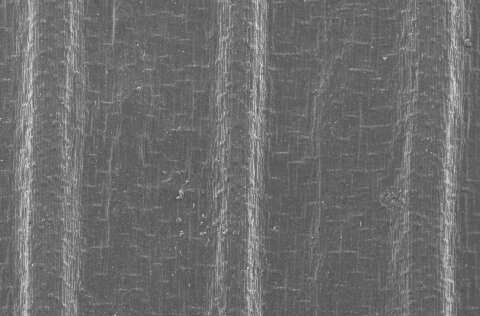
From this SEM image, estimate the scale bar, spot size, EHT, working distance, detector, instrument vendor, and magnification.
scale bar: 200000 nm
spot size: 3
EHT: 10 kV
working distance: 20.4 mm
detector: SE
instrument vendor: FEI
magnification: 0.1 K X